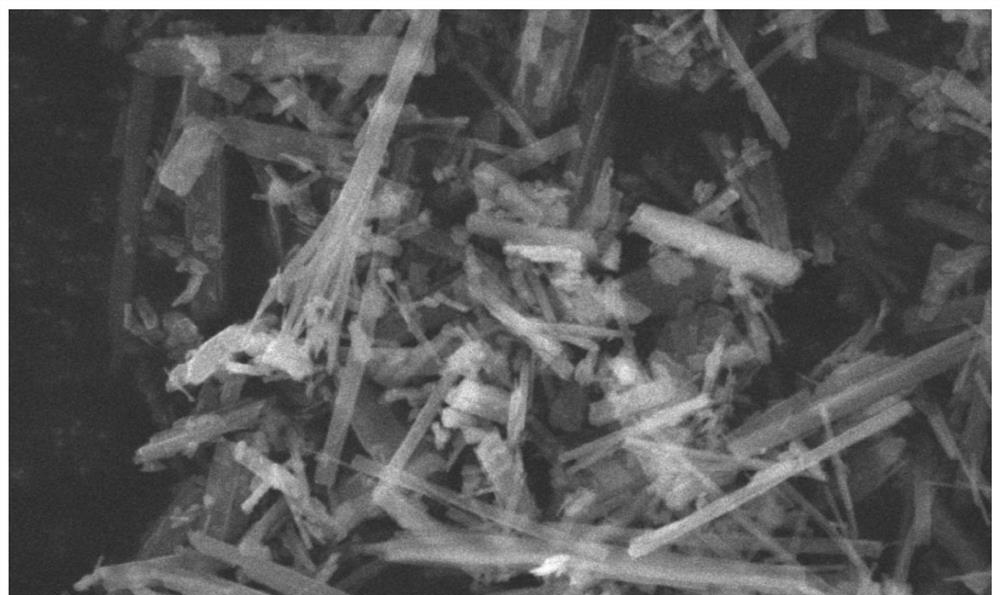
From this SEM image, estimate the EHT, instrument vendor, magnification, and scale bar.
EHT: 20 kV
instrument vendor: JEOL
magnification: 10 K X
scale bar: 1000 nm